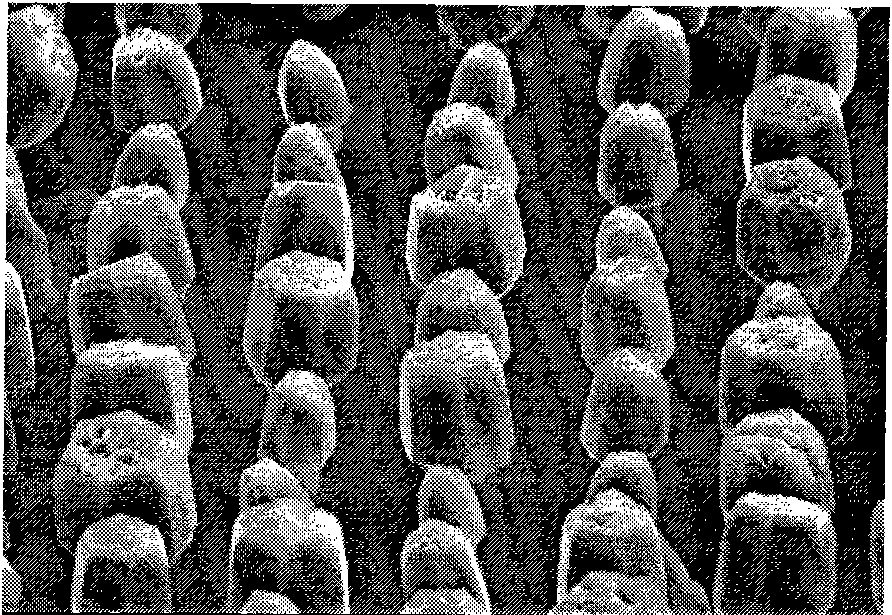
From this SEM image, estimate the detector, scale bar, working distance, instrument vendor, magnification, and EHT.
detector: SE(M)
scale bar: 5000 nm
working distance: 13.4 mm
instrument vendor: Hitachi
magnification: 6 K X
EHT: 15 kV